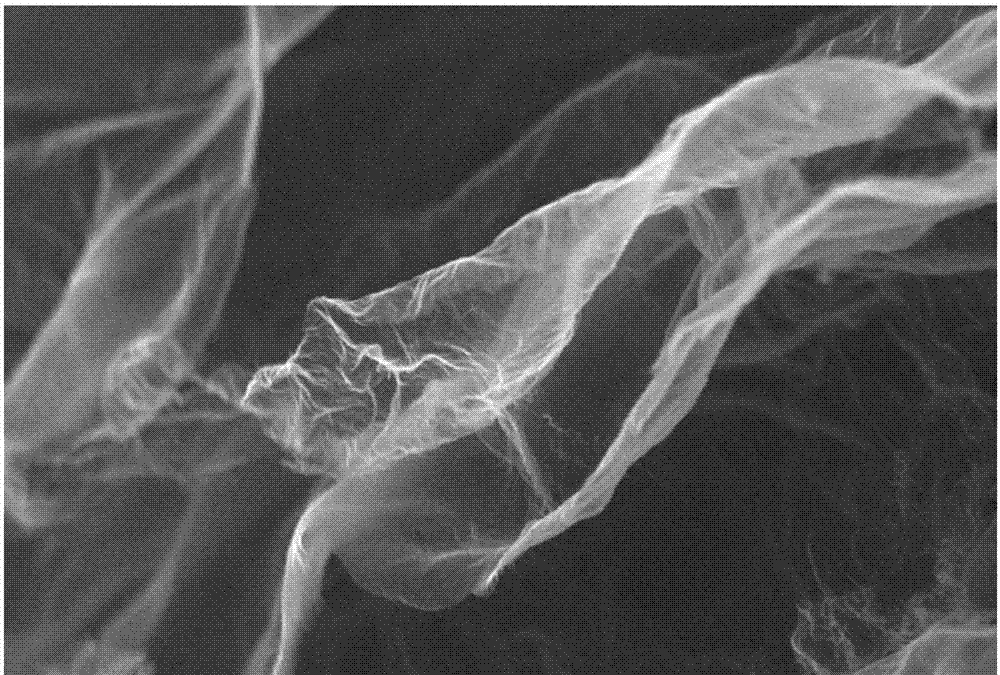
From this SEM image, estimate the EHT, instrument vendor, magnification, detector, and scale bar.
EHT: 20 kV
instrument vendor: JEOL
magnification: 5 K X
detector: SEI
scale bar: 5000 nm